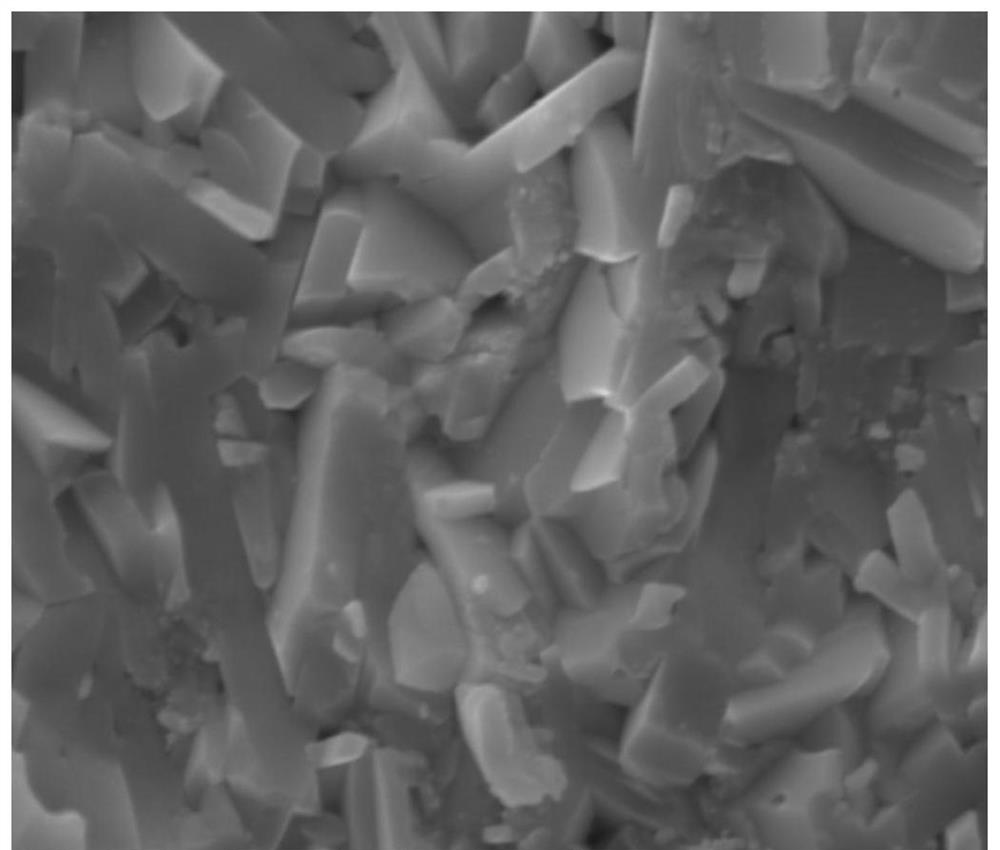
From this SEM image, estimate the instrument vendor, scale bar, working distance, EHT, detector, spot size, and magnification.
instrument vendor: FEI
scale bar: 1000 nm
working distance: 5.6 mm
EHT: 18 kV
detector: ETD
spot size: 4.5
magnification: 50 K X